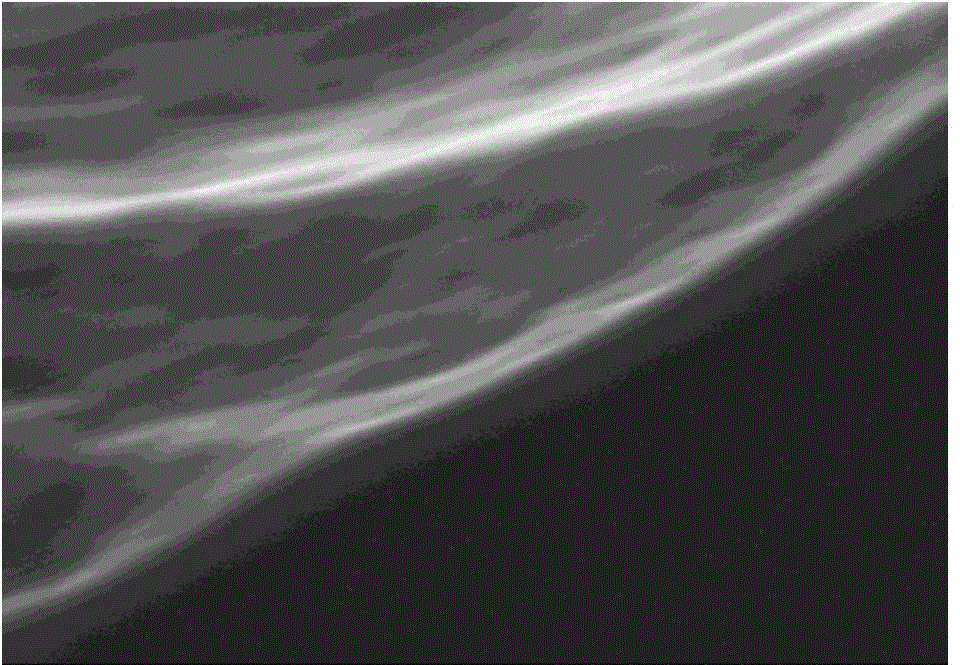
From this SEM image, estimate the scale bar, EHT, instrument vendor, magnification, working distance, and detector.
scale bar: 500 nm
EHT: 5 kV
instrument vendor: Hitachi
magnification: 100 K X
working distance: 5.8 mm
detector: SE(M)